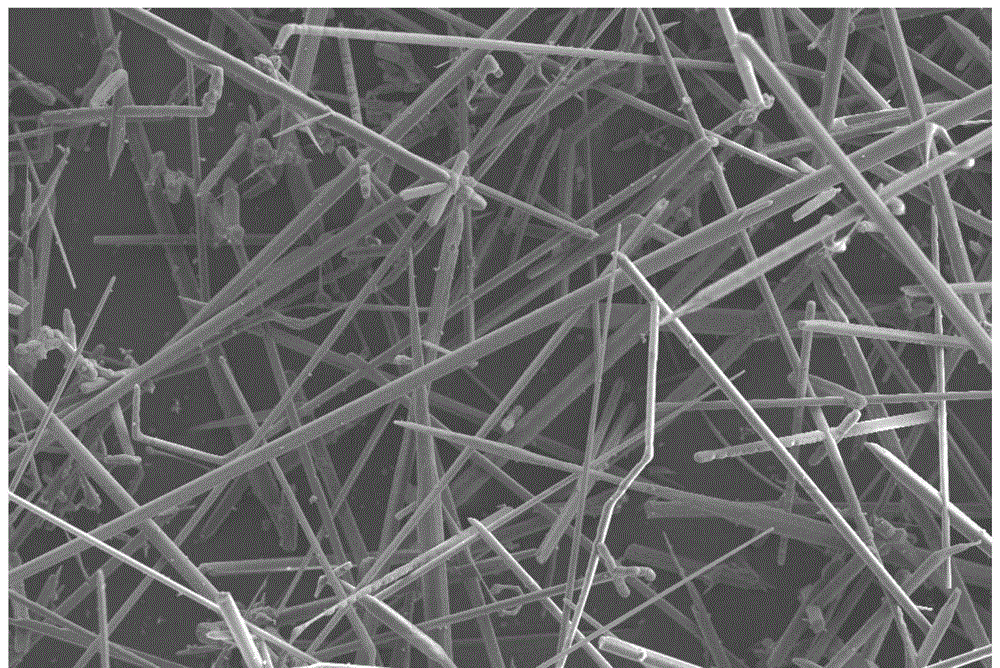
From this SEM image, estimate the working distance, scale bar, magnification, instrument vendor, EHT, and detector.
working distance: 6.7 mm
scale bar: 20000 nm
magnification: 0.5 K X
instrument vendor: Zeiss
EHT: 10 kV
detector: SE2